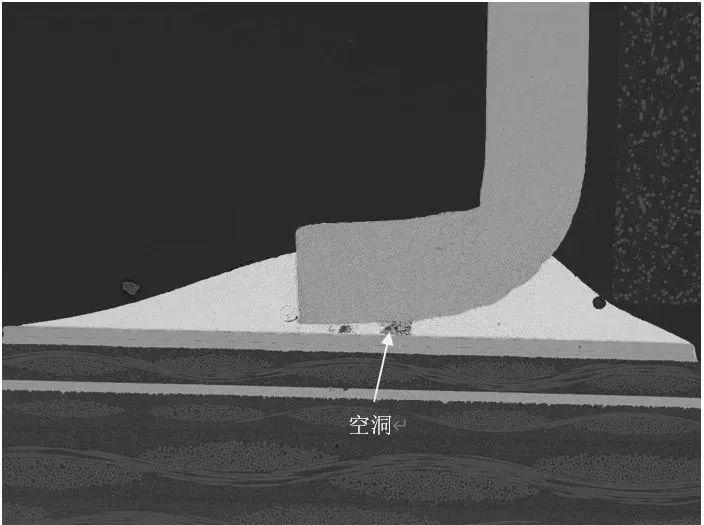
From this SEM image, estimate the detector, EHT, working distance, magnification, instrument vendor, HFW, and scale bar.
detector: BSE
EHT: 20 kV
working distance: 12 mm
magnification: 0.136 K X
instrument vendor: Tescan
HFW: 2040 µm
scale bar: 500000 nm